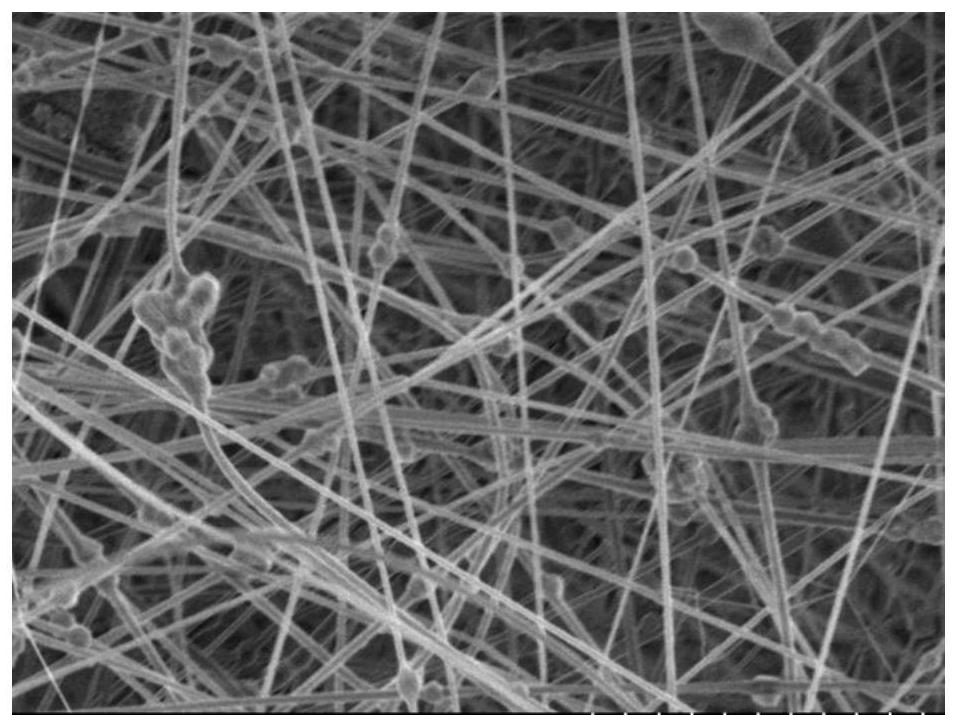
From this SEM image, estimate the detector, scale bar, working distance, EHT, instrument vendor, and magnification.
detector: SE(UL)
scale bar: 10000 nm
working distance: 8.7 mm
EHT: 3 kV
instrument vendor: Hitachi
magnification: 4.5 K X